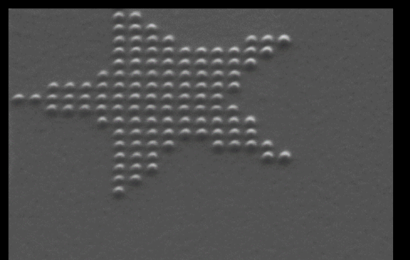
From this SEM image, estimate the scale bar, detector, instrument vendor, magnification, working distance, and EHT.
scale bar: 100 nm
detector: SE2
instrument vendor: Zeiss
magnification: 141.12 K X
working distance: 12.2 mm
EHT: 20 kV